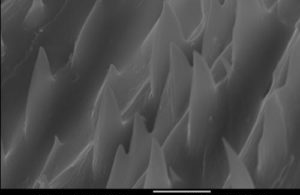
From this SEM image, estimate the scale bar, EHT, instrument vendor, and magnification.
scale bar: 5000 nm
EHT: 15 kV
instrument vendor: JEOL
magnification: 5 K X